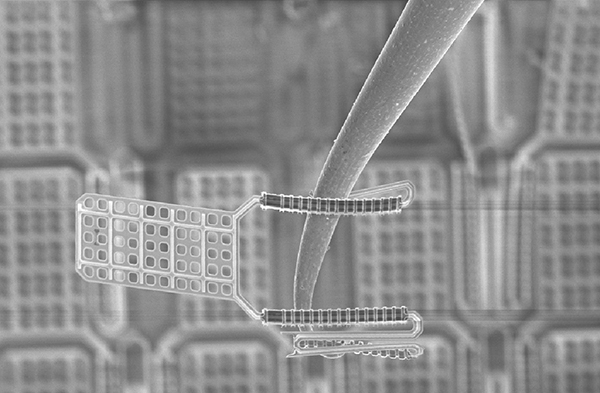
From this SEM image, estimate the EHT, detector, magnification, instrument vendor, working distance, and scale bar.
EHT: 5 kV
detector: InLens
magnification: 2.24 K X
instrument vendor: Zeiss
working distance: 9 mm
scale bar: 10000 nm